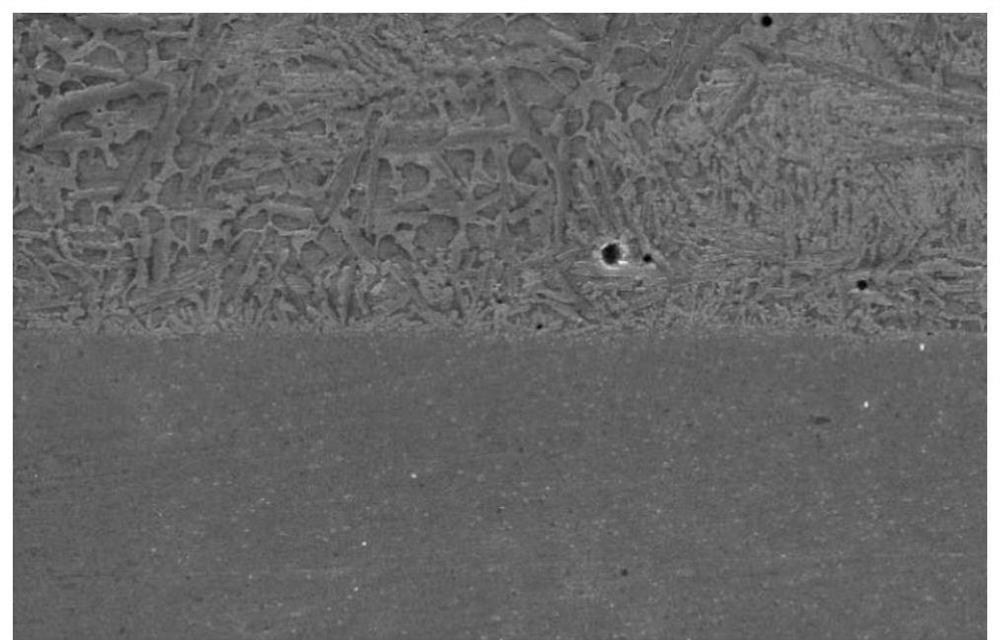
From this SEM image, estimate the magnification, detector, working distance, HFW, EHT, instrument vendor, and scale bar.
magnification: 2 K X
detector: SE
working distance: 14.14 mm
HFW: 104 µm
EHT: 30 kV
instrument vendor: Tescan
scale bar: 20000 nm